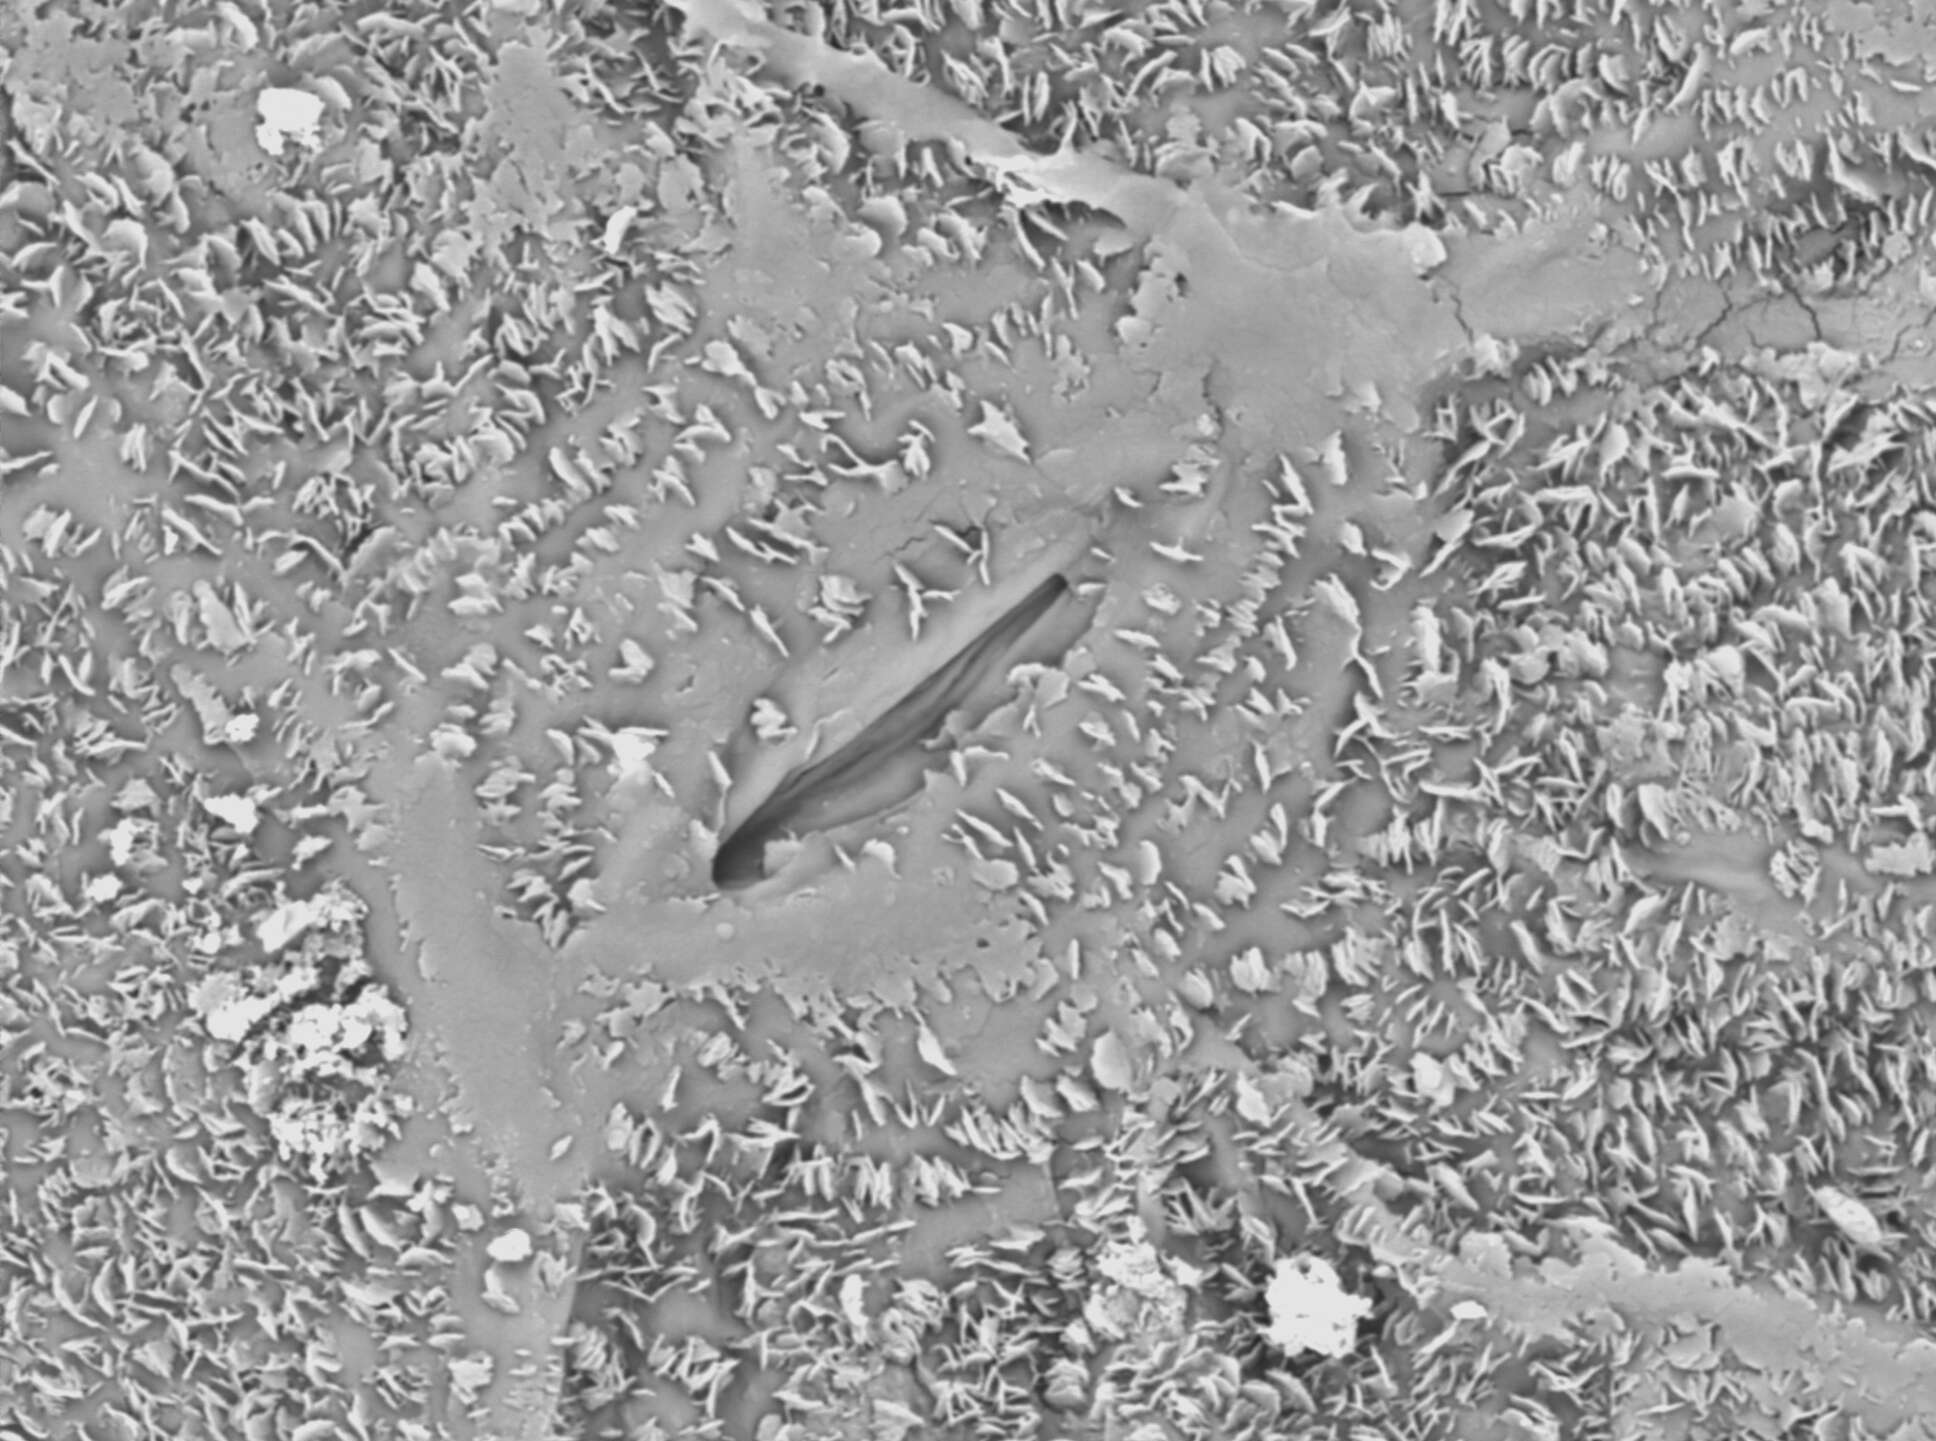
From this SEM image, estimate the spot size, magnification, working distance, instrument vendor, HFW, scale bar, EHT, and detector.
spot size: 4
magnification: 2.5 K X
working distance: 9.7 mm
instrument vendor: FEI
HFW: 49.6 µm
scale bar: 10000 nm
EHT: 10 kV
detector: BSE